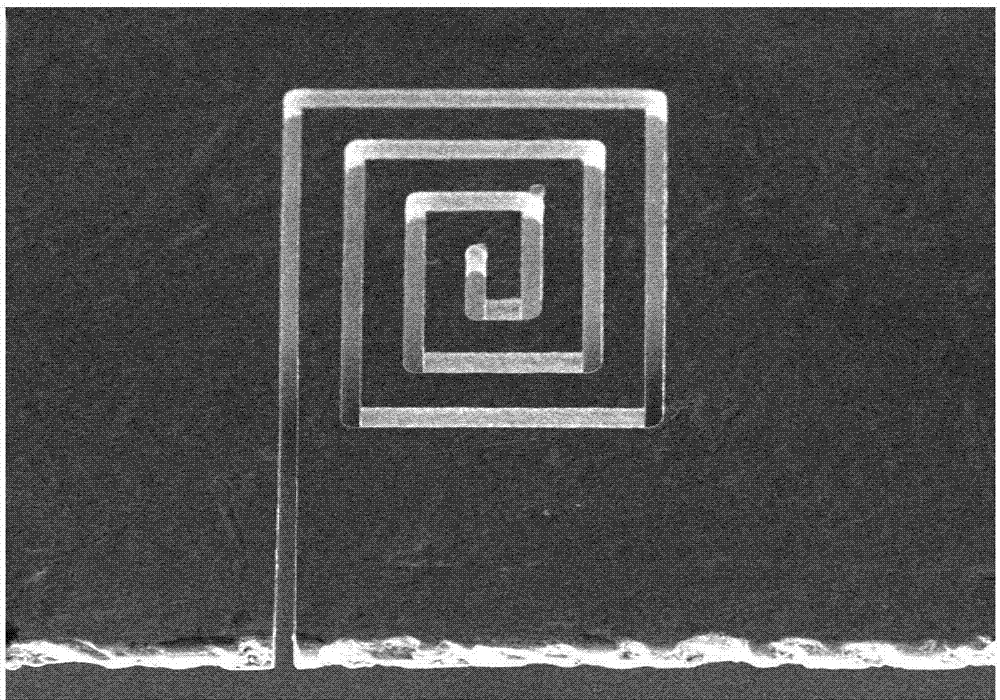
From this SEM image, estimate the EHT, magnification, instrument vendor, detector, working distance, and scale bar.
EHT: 20 kV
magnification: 0.2 K X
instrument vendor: Hitachi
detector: SE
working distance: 9.6 mm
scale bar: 200000 nm